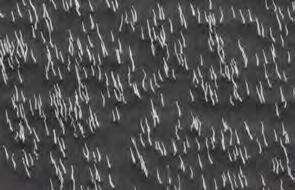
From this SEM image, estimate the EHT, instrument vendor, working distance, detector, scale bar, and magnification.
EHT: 6 kV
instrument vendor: Zeiss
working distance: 6.5 mm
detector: SE2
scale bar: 1000 nm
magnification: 30.03 K X